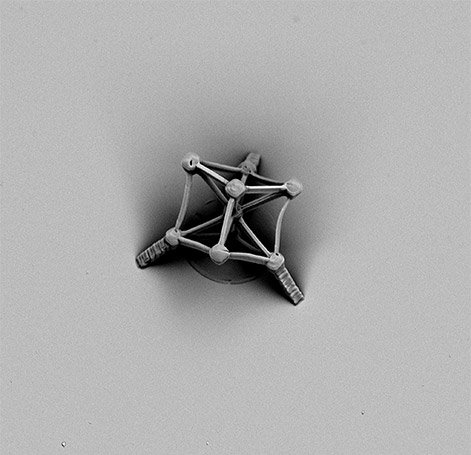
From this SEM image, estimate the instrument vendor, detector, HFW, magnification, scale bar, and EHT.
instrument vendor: other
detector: -Image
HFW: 113 µm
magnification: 2.4 K X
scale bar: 30000 nm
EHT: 10 kV